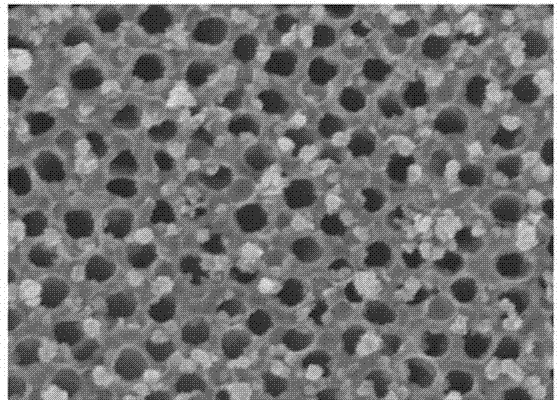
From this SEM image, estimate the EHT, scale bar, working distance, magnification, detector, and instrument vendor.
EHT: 10 kV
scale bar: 500 nm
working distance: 8.1 mm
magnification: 100 K X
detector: SE(M)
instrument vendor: Hitachi